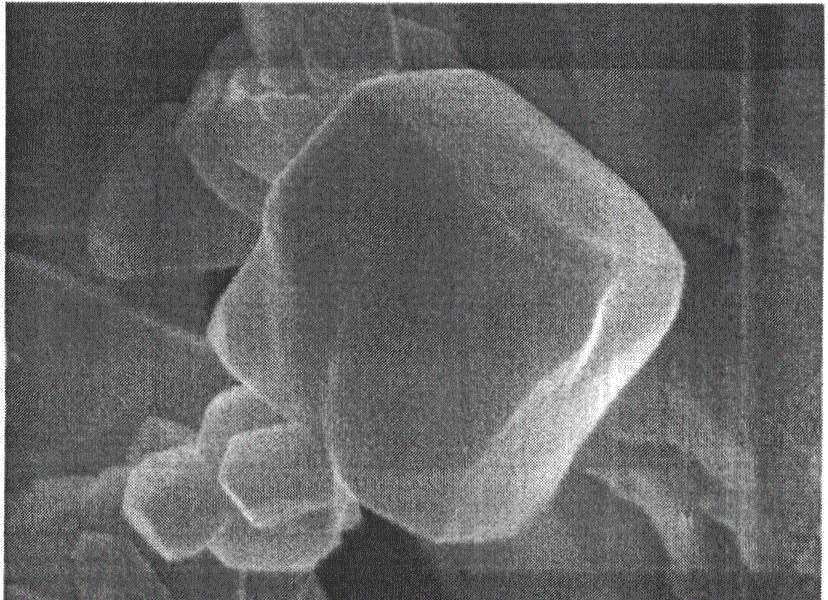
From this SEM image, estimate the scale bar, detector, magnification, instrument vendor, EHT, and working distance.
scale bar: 100 nm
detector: SEI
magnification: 90 K X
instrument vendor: JEOL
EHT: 5 kV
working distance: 7.9 mm